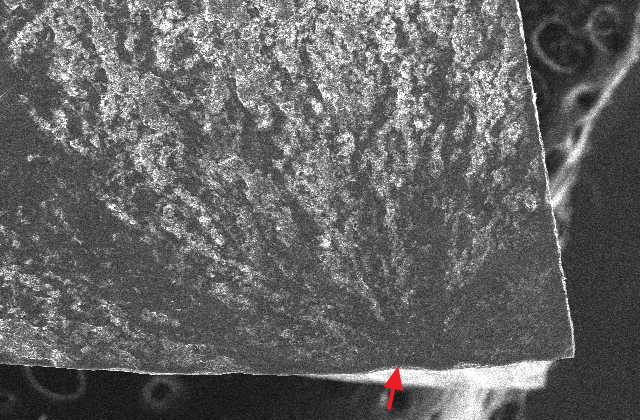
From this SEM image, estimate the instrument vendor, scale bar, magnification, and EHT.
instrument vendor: JEOL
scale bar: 500000 nm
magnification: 0.05 K X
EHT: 20 kV